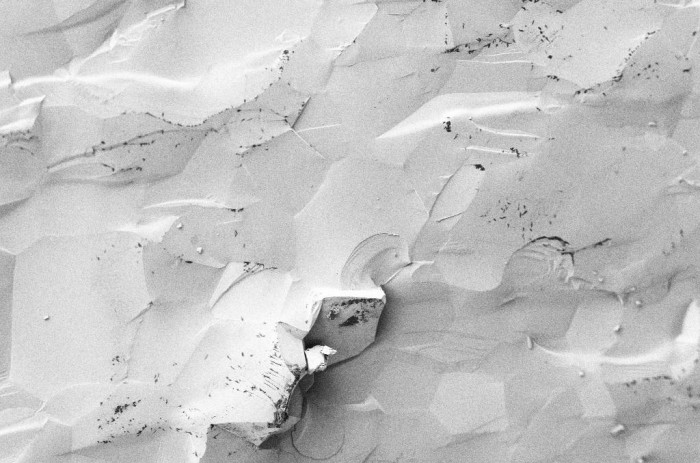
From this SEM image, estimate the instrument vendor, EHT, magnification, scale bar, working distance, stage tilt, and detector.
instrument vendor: Zeiss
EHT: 1 kV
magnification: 1 K X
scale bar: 10000 nm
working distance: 5.4 mm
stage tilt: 36°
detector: SE2